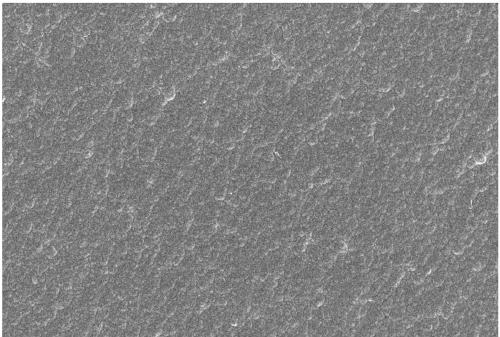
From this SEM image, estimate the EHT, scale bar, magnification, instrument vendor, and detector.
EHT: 15 kV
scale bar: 50000 nm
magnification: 0.5 K X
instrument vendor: JEOL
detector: SEI